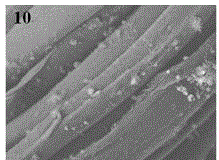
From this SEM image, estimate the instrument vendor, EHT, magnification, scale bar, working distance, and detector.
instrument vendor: JEOL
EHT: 10 kV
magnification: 2 K X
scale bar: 10000 nm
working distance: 8.3 mm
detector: LEI